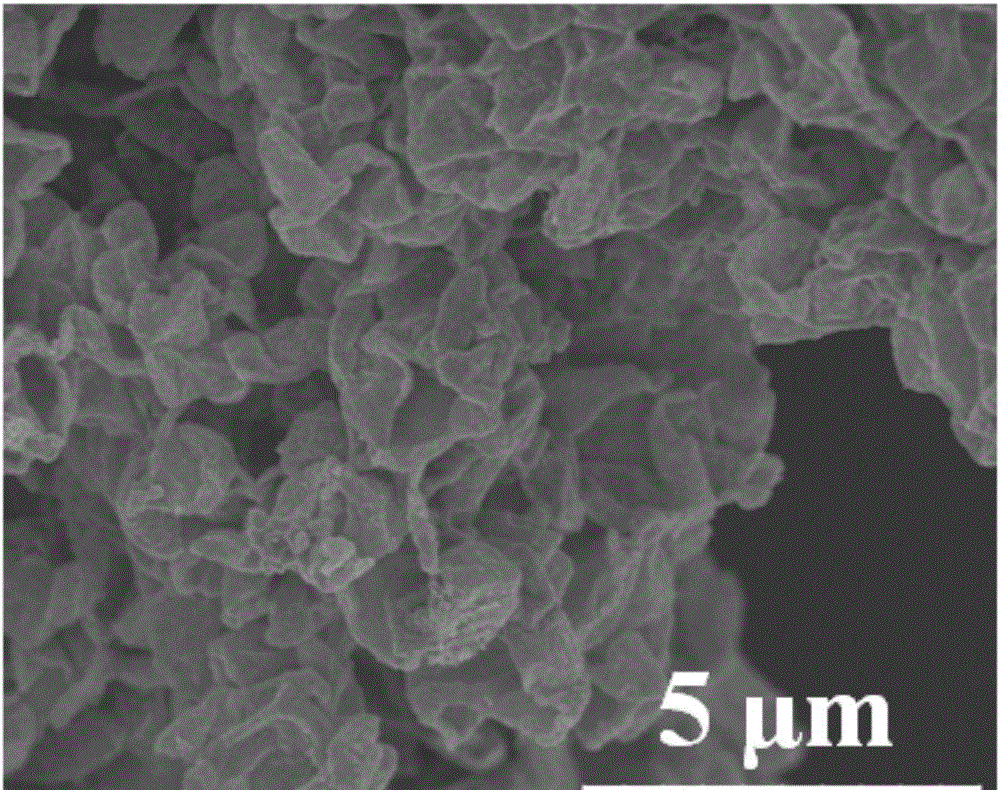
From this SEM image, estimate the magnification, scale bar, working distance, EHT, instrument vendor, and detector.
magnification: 9 K X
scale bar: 5000 nm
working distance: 10.6 mm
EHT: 5 kV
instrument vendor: Hitachi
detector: SE(M)